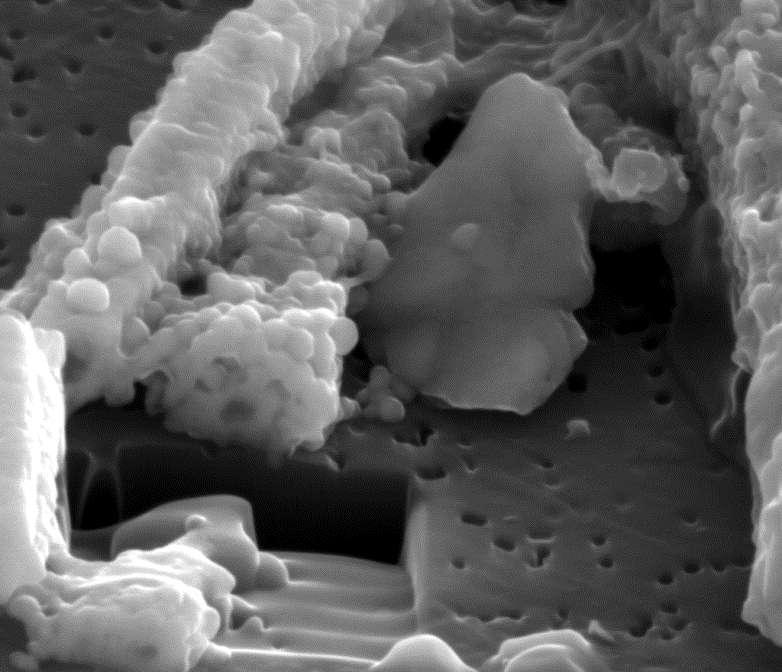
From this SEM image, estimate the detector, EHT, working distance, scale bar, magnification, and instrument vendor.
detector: ETD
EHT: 10 kV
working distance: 10 mm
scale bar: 2000 nm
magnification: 25 K X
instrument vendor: FEI